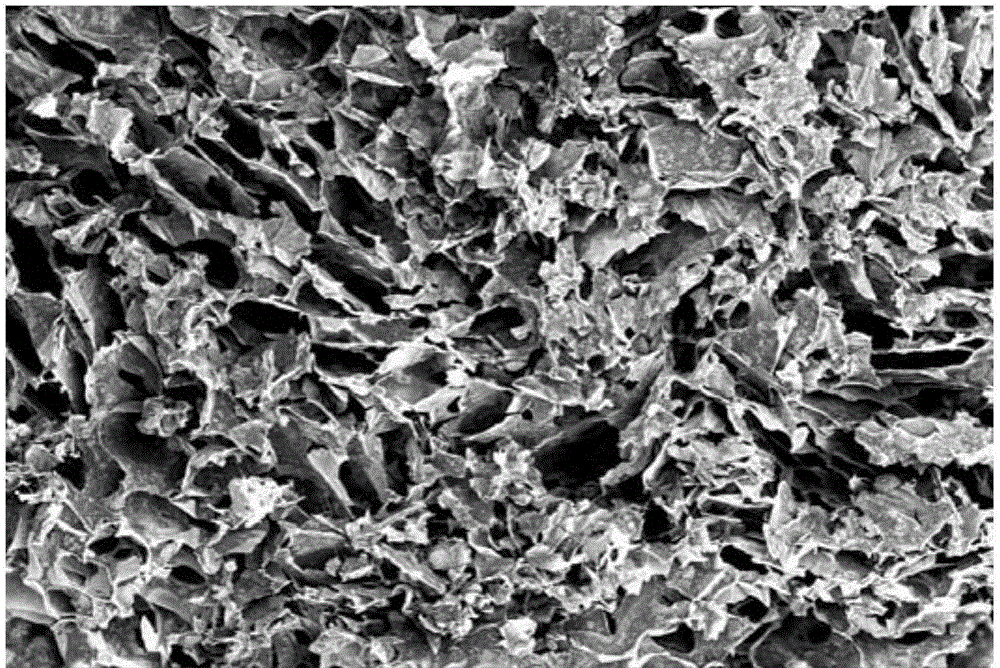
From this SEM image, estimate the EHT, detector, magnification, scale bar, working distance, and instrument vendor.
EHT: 10 kV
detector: SE1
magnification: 0.03 K X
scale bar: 200000 nm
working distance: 12.5 mm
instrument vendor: Zeiss